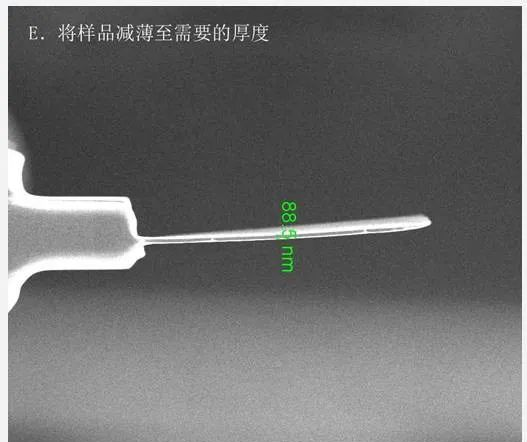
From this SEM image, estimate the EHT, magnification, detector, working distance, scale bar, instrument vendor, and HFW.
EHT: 15 kV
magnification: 24 K X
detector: TLD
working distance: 4.2 mm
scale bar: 4000 nm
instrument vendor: FEI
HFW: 10.7 µm